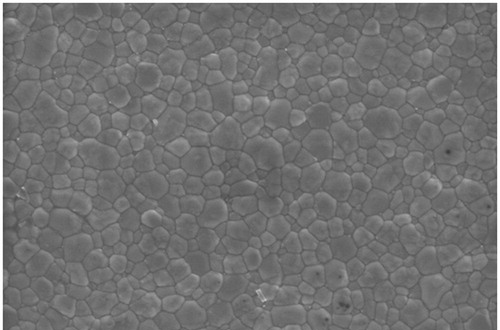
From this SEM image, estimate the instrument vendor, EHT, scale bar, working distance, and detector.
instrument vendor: Zeiss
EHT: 5 kV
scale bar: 1000 nm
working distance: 2.9 mm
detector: InLens